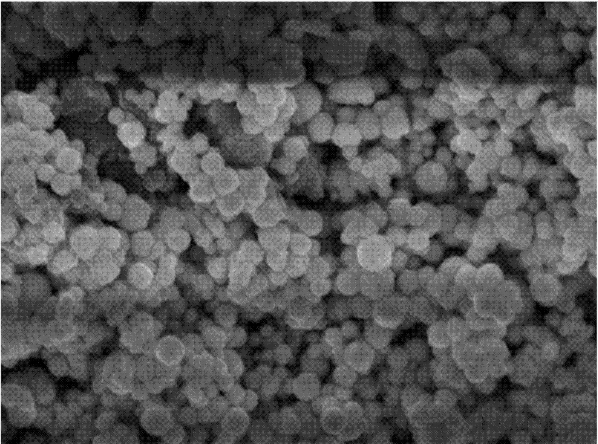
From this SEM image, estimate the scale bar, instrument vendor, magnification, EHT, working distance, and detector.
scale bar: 100 nm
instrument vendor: JEOL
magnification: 30 K X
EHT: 8 kV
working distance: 7.5 mm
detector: SEI